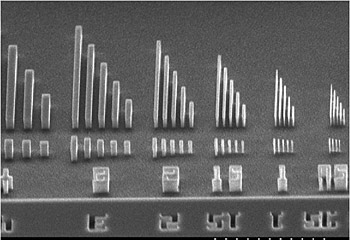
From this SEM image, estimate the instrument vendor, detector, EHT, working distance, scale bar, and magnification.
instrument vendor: JEOL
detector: SE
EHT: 20 kV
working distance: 28 mm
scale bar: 50000 nm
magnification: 0.8 K X